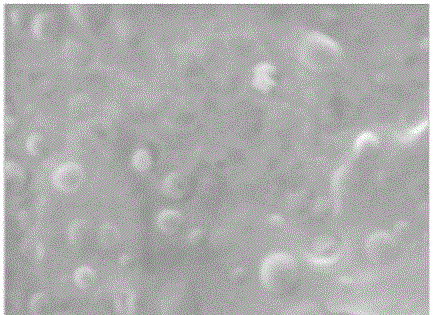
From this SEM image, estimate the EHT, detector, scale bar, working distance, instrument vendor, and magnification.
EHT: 15 kV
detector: SE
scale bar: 2000 nm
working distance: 27.19 mm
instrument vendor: Tescan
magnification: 13.2 K X